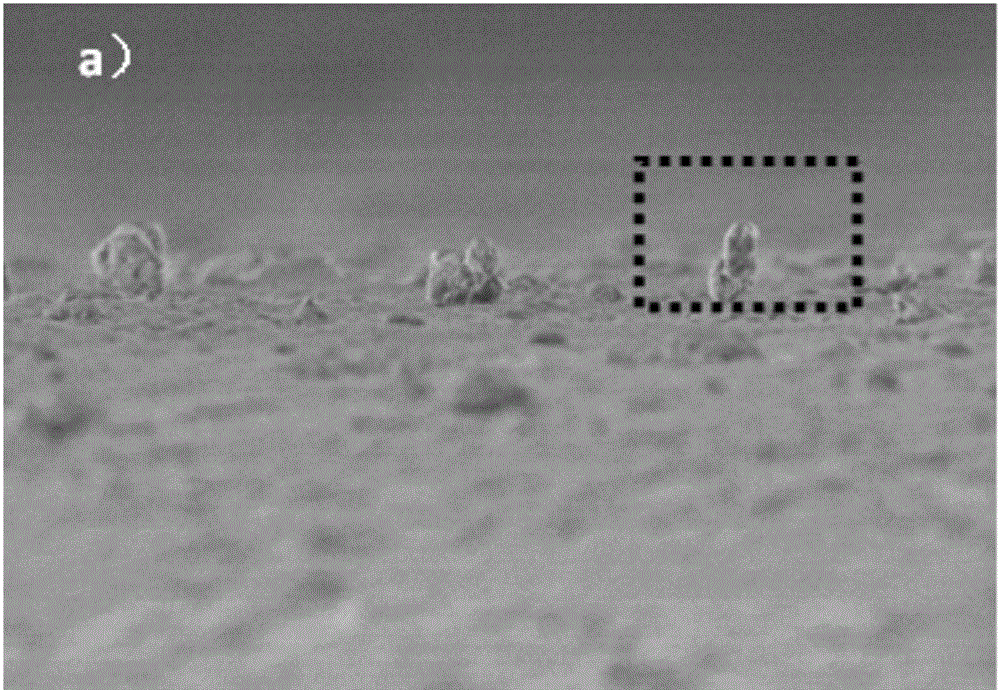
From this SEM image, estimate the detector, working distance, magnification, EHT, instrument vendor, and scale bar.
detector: SE(UL)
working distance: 9.1 mm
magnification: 4.5 K X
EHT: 3 kV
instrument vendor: Hitachi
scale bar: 10000 nm